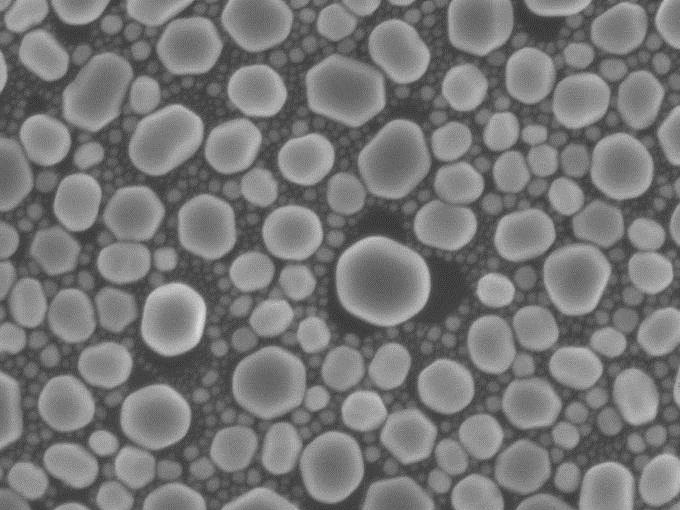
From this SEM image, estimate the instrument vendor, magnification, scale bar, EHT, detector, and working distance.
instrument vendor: JEOL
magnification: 50 K X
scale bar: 100 nm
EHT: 5 kV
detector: SEI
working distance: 6 mm